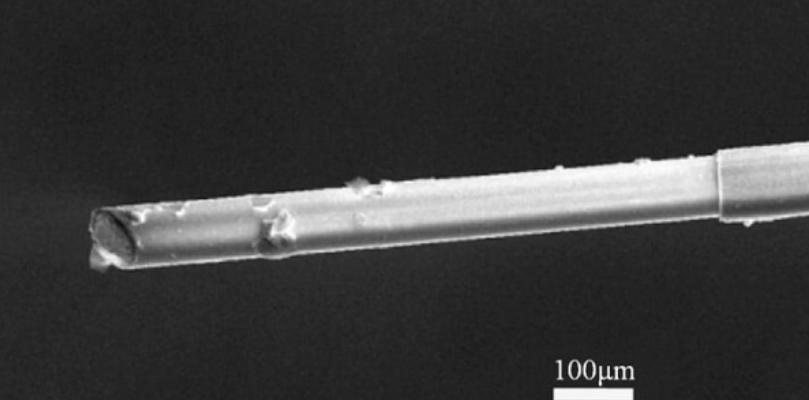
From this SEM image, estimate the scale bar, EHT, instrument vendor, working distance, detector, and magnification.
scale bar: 100000 nm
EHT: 20 kV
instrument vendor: FEI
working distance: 14.2 mm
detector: SE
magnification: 0.5 K X